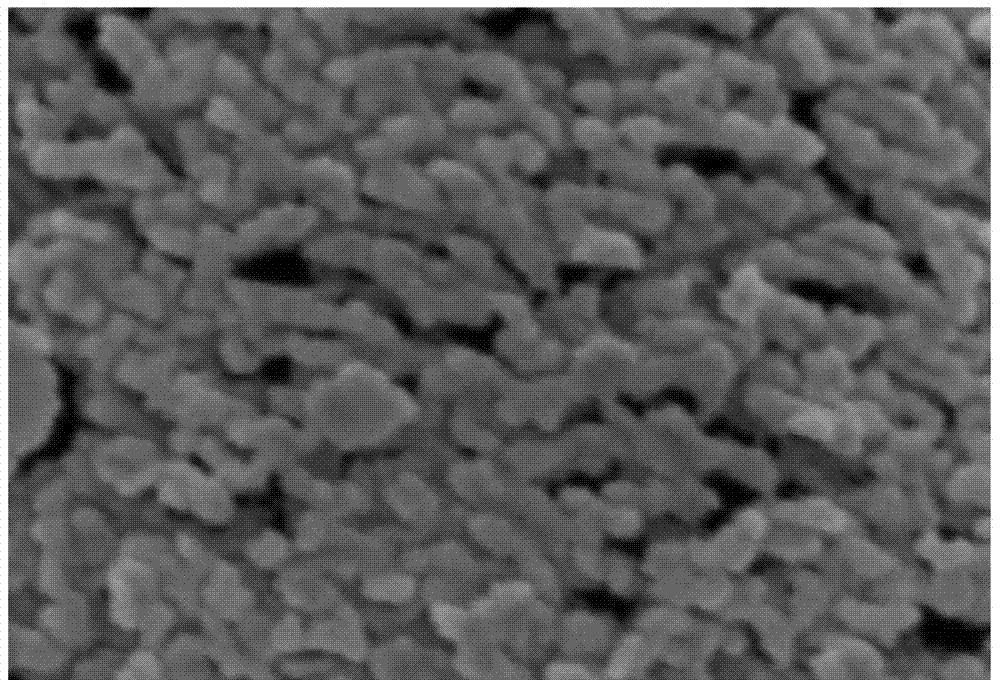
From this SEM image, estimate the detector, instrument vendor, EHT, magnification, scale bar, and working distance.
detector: SE(U)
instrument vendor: Hitachi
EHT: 3 kV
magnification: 100 K X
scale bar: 500 nm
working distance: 9.1 mm